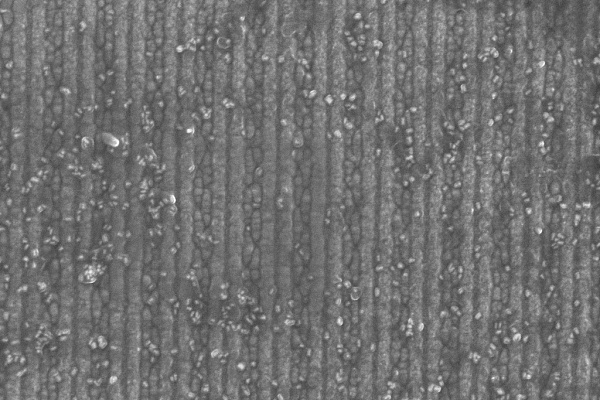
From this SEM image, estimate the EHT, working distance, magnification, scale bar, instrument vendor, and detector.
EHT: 10 kV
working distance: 5.3 mm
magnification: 100 K X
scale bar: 200 nm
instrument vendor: Zeiss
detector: InLens